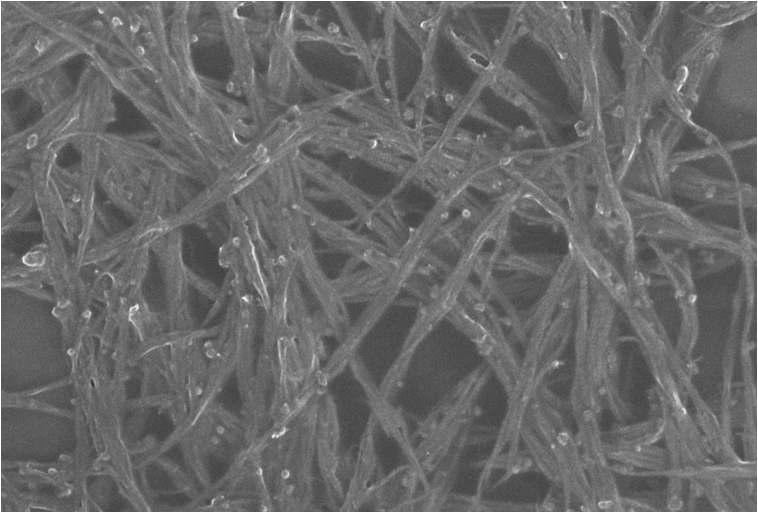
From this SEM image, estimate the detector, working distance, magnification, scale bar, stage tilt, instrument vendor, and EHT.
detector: InLens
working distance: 4.3 mm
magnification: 100 K X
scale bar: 100 nm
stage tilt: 57.5°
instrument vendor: Zeiss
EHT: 10 kV